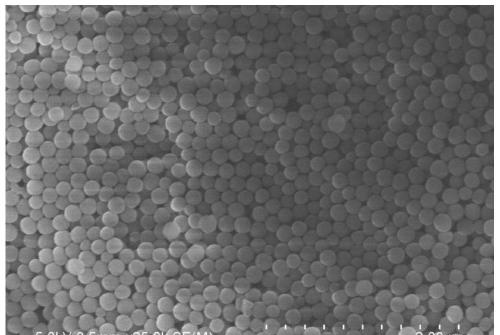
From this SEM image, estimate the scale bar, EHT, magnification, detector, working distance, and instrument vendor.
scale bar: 2000 nm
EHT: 5 kV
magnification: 25 K X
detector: SE(M)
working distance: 8.5 mm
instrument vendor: Hitachi